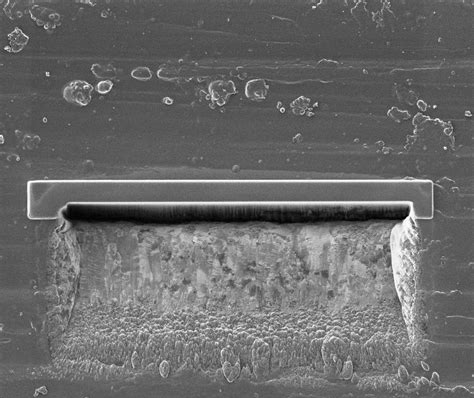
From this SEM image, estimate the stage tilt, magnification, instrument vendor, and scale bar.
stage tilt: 0°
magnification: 6.5 K X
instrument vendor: FEI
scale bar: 20000 nm